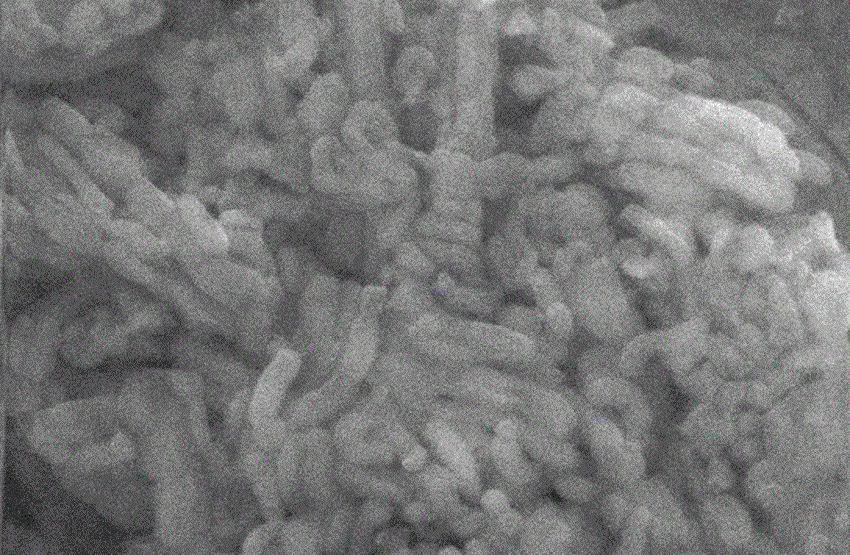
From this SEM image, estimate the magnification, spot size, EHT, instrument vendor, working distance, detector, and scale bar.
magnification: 10 K X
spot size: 5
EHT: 20 kV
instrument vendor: FEI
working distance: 5.9 mm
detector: SE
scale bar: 2000 nm